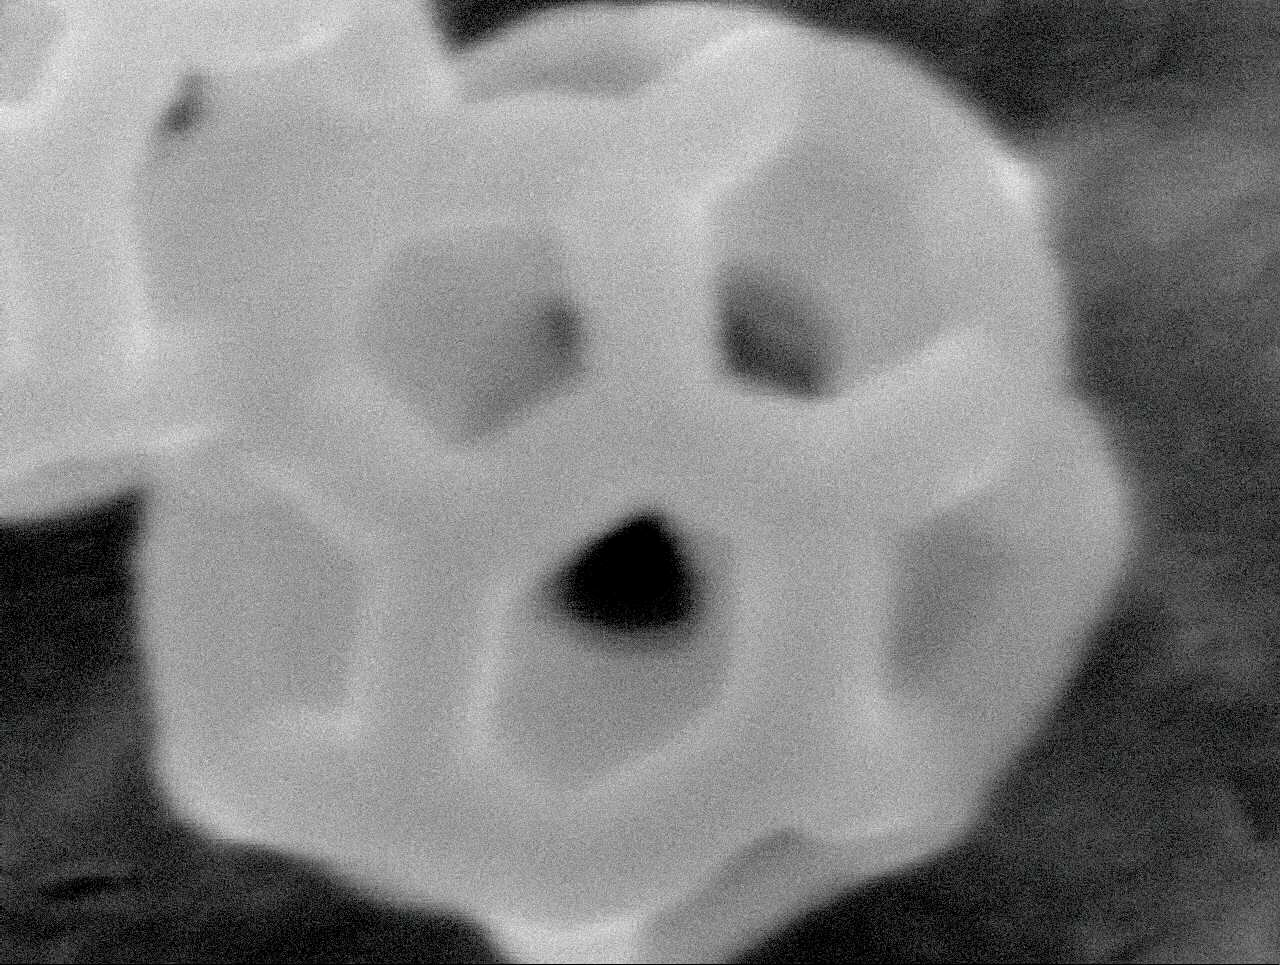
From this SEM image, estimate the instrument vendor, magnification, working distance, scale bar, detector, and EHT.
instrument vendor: JEOL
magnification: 250 K X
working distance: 10.1 mm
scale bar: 100 nm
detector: SEI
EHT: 5 kV